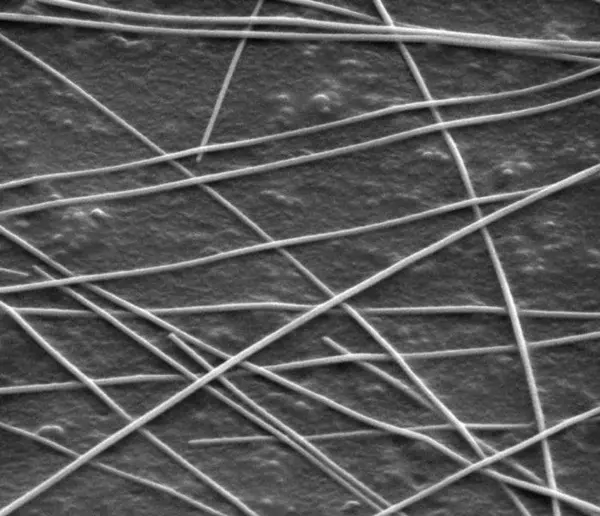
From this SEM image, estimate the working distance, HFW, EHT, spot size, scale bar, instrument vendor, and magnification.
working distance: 7.5 mm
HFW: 2.98 µm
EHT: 5 kV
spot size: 4.5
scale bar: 1000 nm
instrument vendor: FEI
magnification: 50 K X